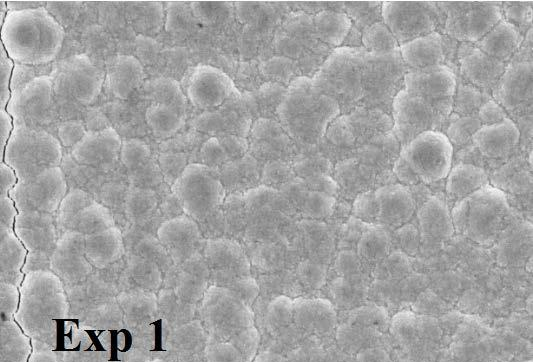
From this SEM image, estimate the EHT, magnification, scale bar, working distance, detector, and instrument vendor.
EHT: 20 kV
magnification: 5 K X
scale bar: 2000 nm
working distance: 25 mm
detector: SE1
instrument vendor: Zeiss